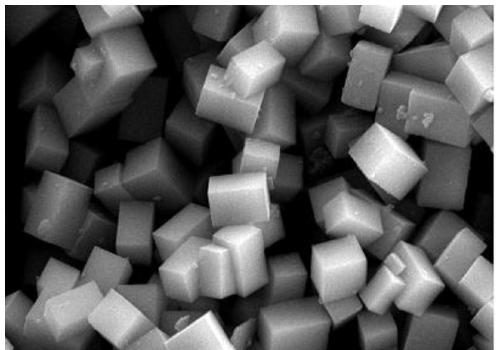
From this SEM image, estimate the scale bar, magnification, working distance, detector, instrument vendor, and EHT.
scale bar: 200 nm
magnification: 50 K X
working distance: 6.4 mm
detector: SE2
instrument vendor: Zeiss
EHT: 10 kV